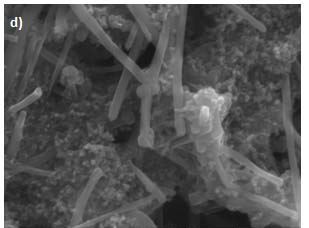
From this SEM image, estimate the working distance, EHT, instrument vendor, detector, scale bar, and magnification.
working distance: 13.9 mm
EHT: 10 kV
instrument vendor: JEOL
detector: SEI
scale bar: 1000 nm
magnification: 20 K X